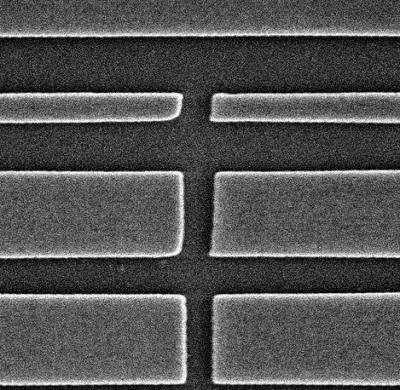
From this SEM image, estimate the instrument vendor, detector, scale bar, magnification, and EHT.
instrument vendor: Tescan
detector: SE Detector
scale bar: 2000 nm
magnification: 25 K X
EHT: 30 kV